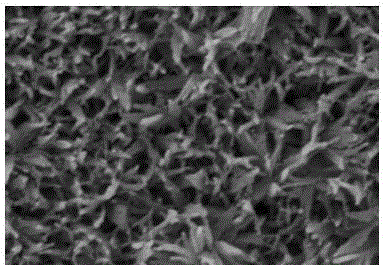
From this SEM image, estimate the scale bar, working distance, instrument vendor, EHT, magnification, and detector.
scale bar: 500 nm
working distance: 6.8 mm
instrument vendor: Hitachi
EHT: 5 kV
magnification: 100 K X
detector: SE(M)